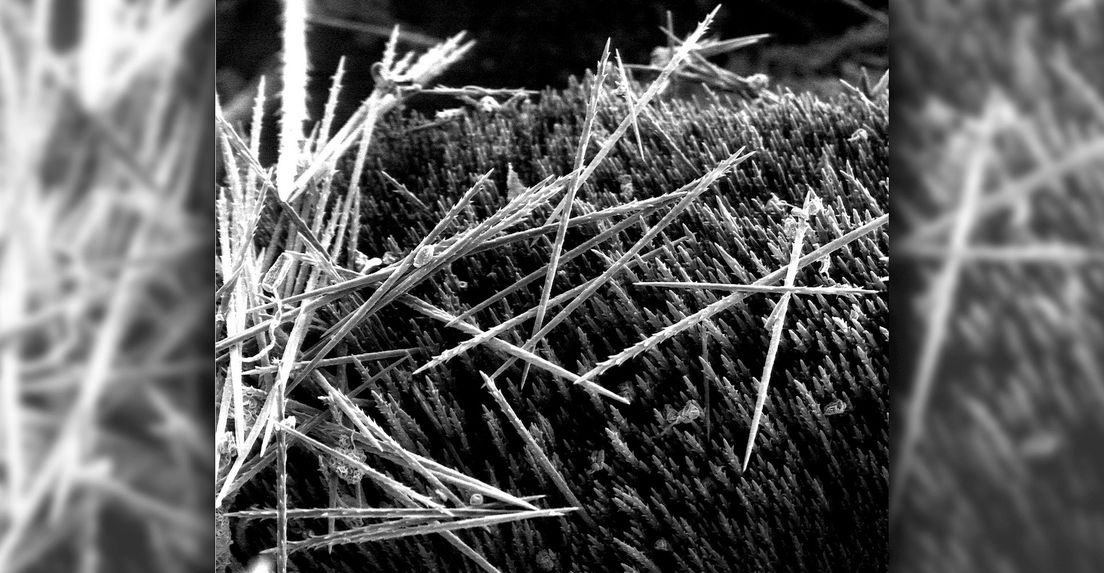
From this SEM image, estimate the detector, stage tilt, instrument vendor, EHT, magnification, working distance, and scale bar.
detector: LFD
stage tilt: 0°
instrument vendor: FEI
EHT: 10 kV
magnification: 0.537 K X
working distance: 12.9 mm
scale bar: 200000 nm